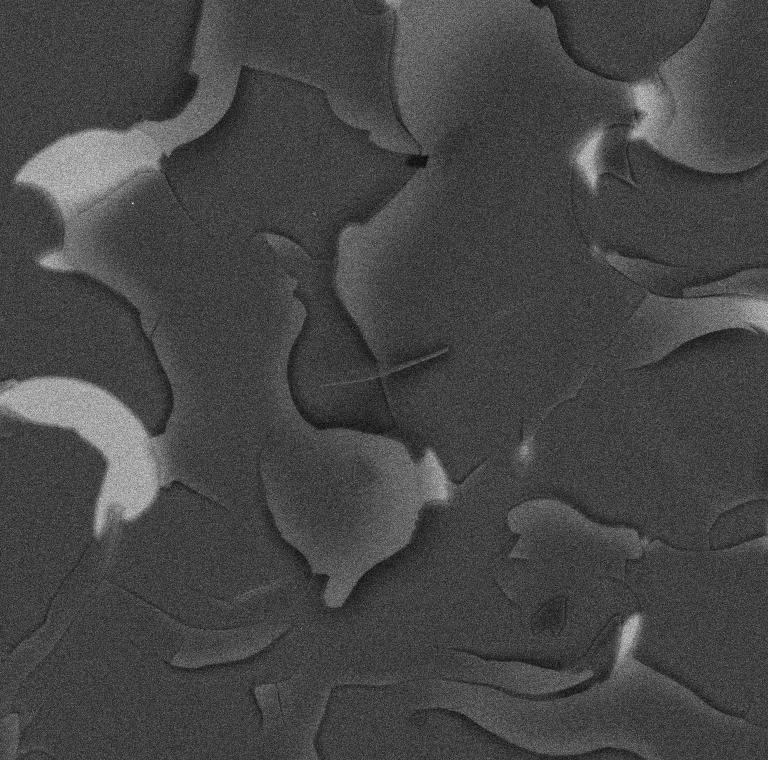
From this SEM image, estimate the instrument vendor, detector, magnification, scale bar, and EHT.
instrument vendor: Tescan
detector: BSE Detector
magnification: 1 K X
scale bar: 100000 nm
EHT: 13 kV